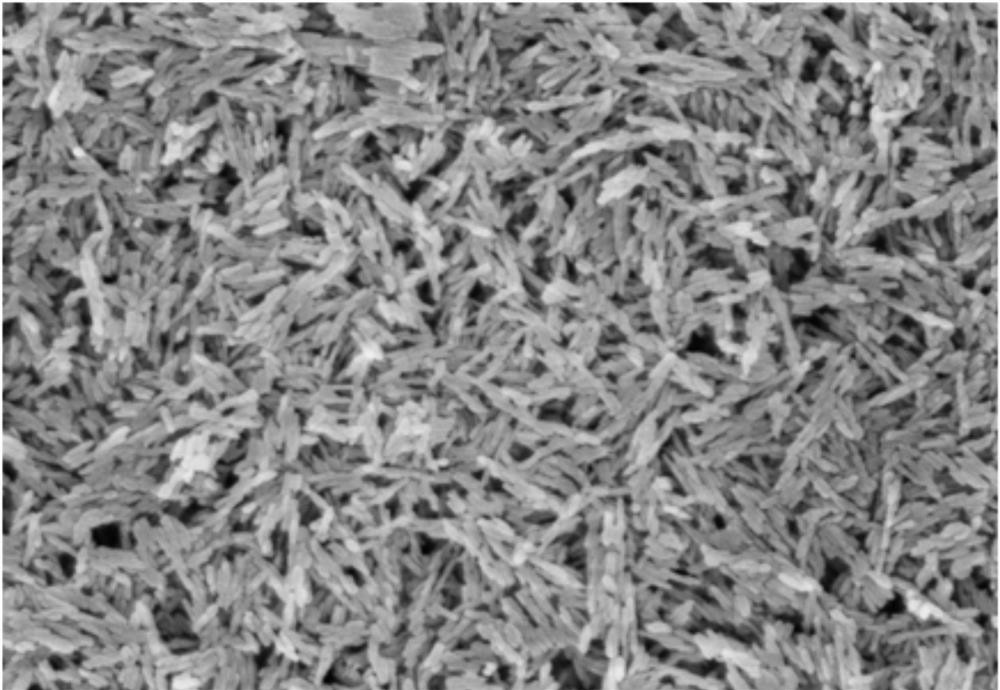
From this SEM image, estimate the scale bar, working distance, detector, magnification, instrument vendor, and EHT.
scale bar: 1000 nm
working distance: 3 mm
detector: SE(TUL)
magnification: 50 K X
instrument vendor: Hitachi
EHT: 3 kV